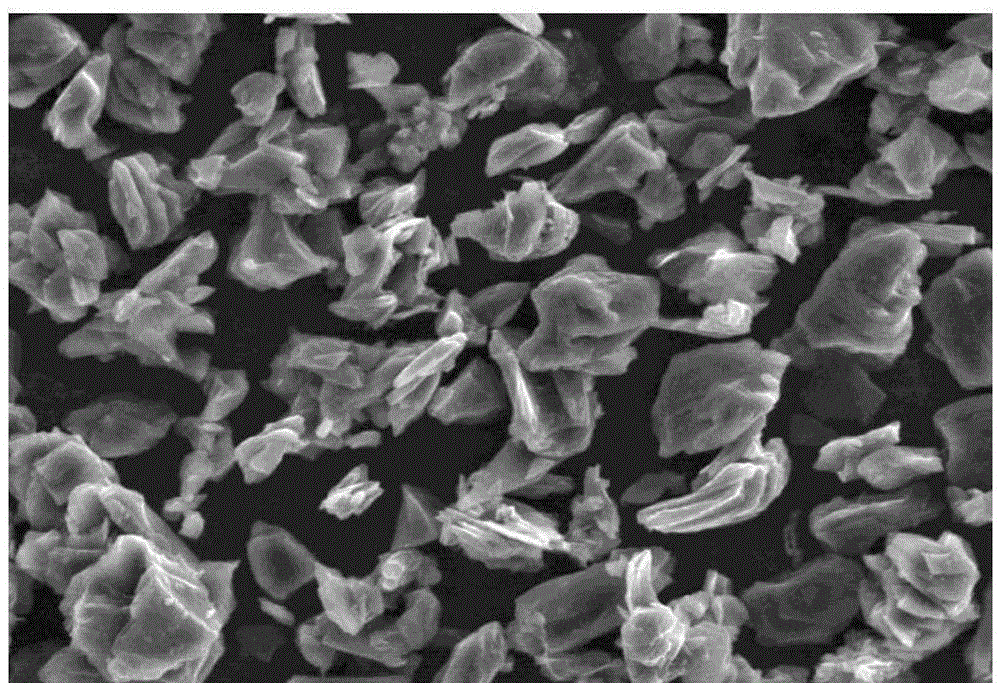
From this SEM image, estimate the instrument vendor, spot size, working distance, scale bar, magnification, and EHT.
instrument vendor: FEI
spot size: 4.5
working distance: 9 mm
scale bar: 50000 nm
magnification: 1 K X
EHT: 20 kV